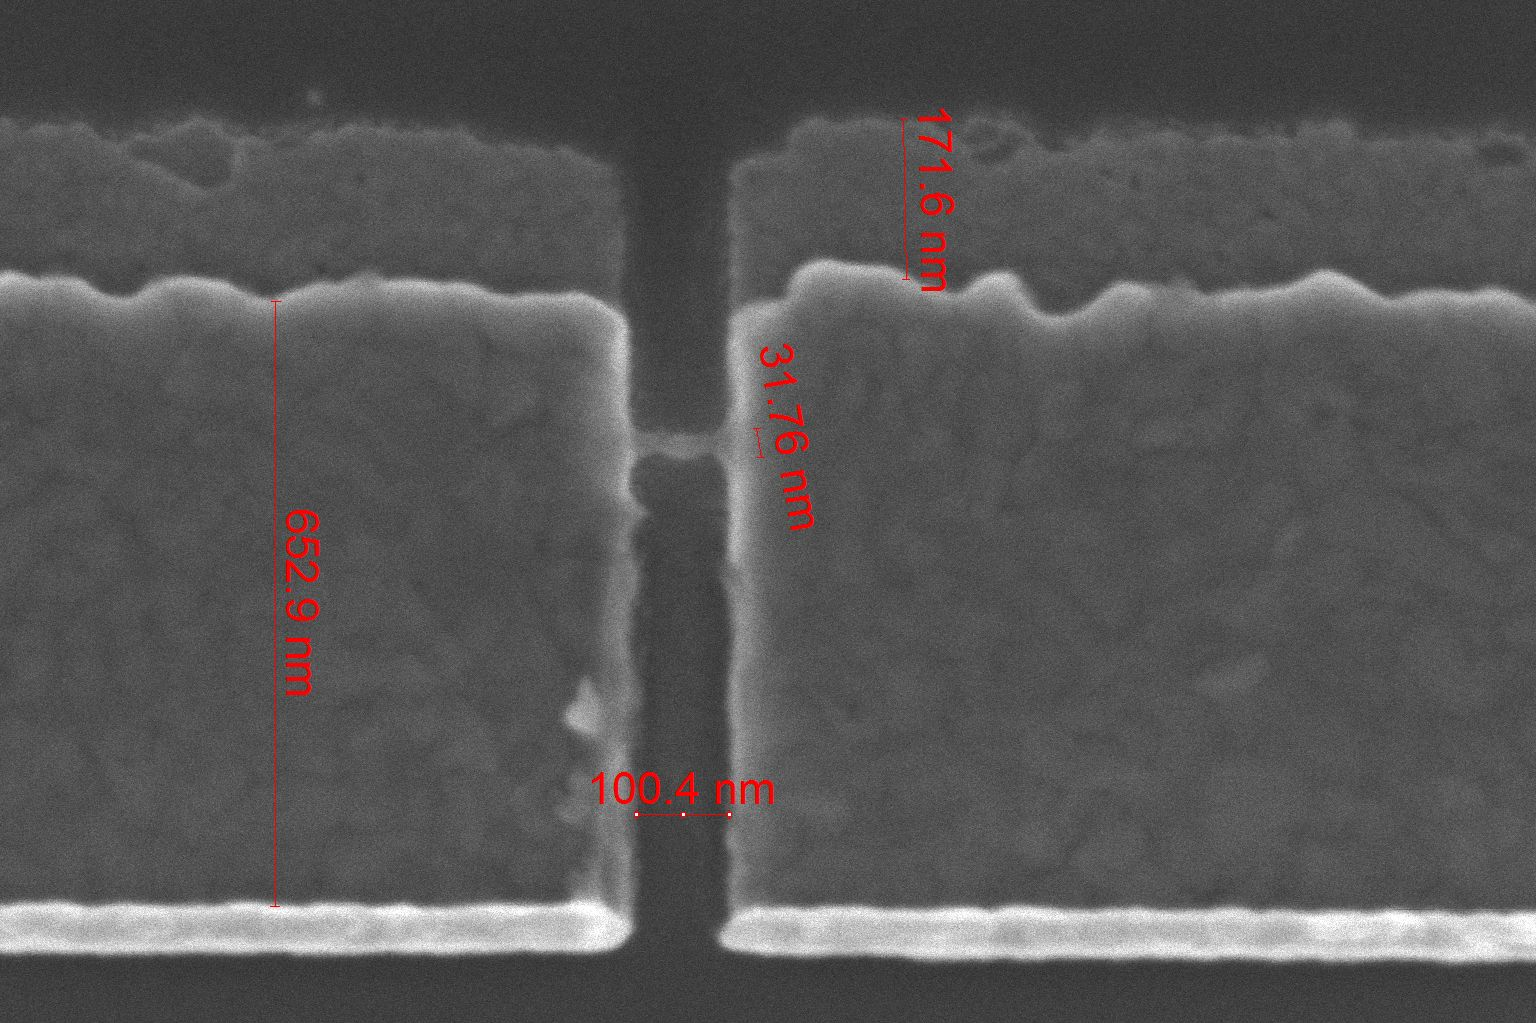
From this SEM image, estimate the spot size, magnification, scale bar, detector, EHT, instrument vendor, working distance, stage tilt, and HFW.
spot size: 2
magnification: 250 K X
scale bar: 400 nm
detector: TLD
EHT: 2 kV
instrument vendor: FEI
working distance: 5.4 mm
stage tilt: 0°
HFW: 1.66 µm